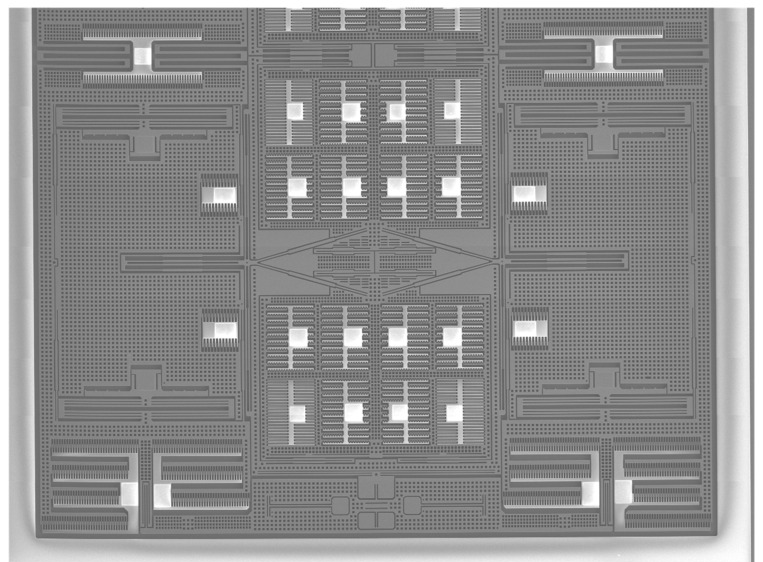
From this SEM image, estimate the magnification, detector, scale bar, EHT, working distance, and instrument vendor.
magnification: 0.033 K X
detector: LED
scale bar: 100000 nm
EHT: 15 kV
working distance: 15 mm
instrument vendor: JEOL